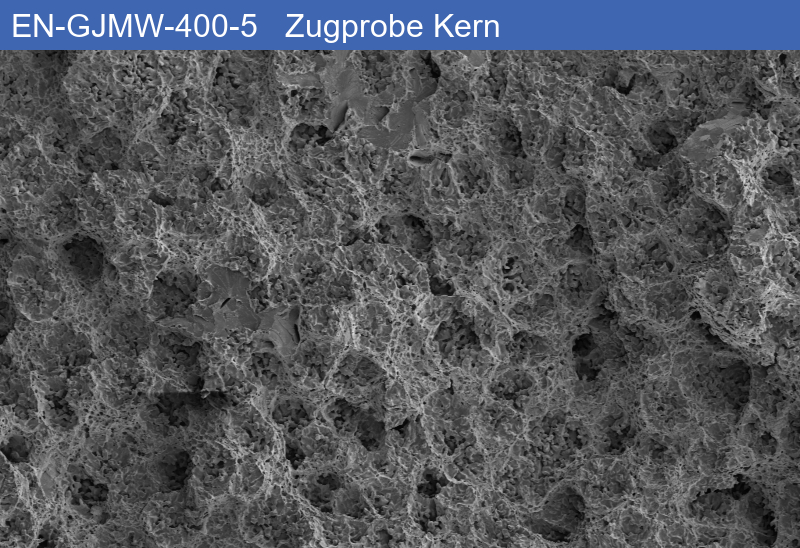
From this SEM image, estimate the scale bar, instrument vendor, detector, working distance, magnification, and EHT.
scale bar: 200000 nm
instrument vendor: Zeiss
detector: SE1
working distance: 10.77 mm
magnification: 0.05 K X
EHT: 10 kV